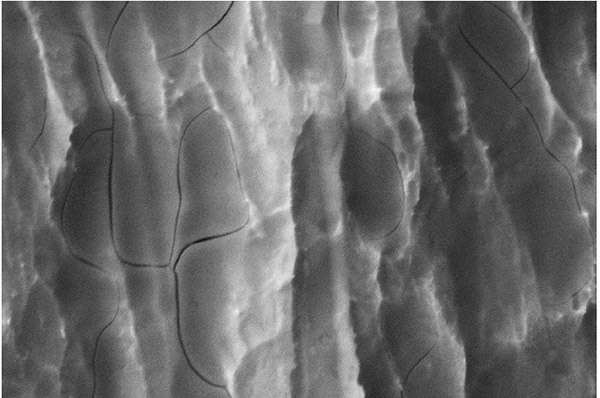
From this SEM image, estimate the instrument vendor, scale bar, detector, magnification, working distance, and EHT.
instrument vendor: Zeiss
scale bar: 2000 nm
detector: SE2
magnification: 10 K X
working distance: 10.6 mm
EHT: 10 kV